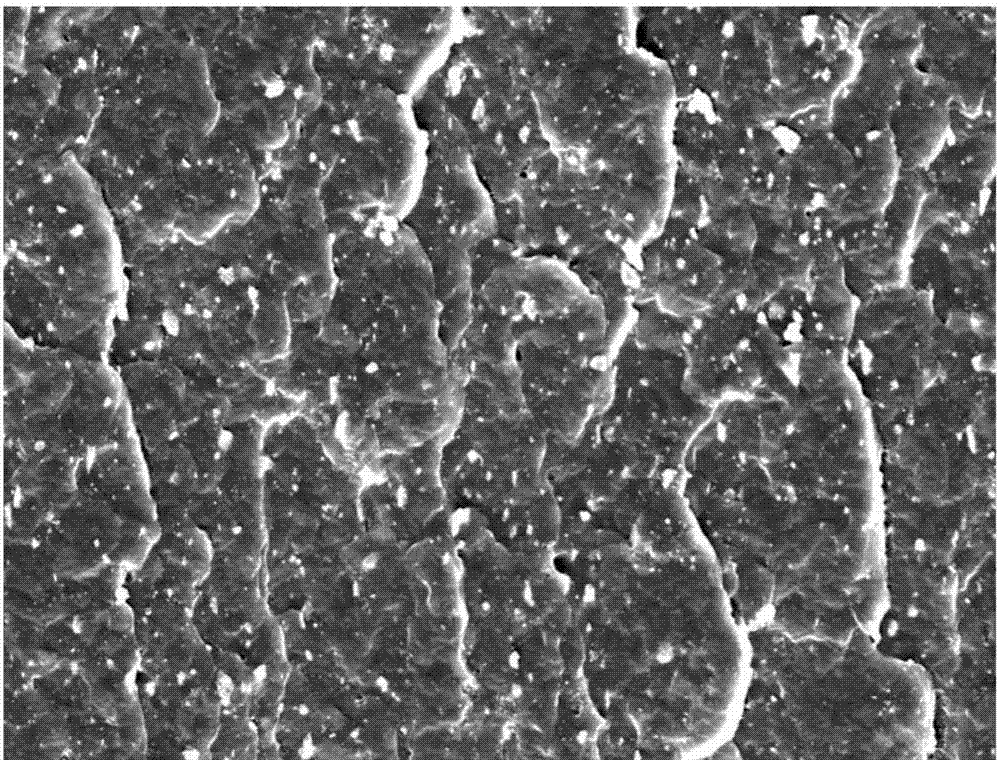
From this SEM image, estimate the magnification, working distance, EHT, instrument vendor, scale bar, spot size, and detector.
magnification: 2 K X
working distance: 5 mm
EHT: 15 kV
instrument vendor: FEI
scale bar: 50000 nm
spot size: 3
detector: ETD